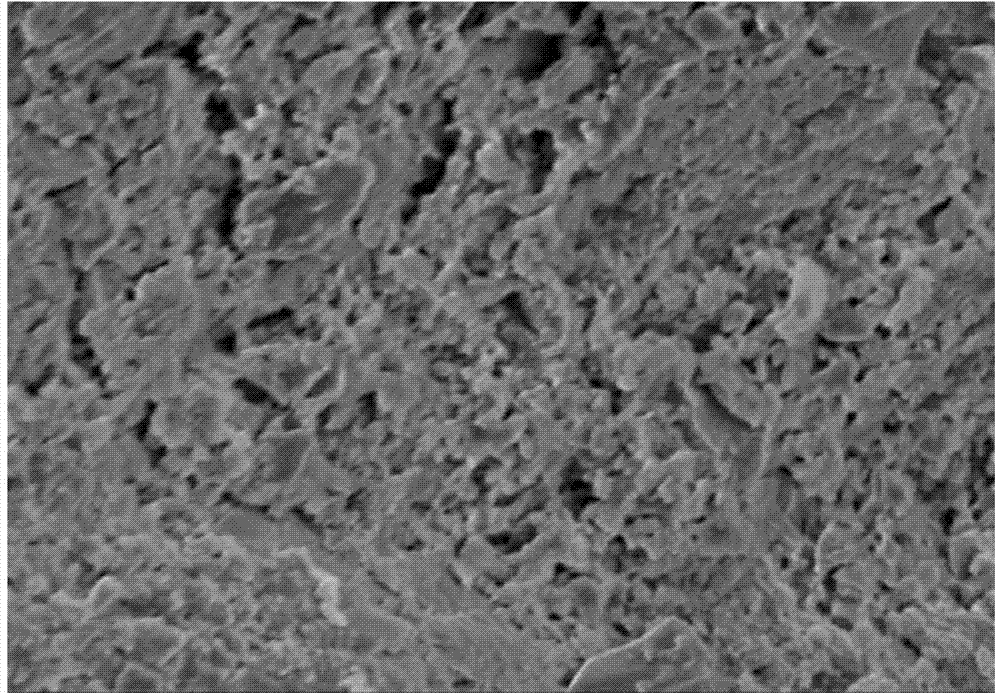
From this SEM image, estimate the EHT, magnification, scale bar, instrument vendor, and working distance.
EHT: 5 kV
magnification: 5 K X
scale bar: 10000 nm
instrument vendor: Hitachi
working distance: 15.7 mm